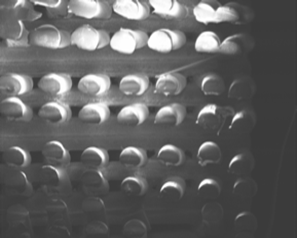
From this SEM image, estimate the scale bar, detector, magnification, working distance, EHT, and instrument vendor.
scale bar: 1000000 nm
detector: SE(M)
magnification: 0.03 K X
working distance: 11.1 mm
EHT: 3 kV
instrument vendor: Hitachi